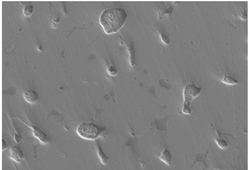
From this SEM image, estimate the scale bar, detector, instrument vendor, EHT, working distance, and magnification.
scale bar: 30000 nm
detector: SE(L)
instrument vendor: Hitachi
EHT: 15 kV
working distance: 6.7 mm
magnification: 1.5 K X